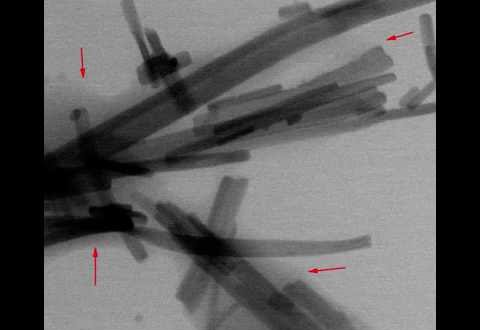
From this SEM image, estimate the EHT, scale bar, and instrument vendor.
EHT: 30 kV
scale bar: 3000 nm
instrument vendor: FEI